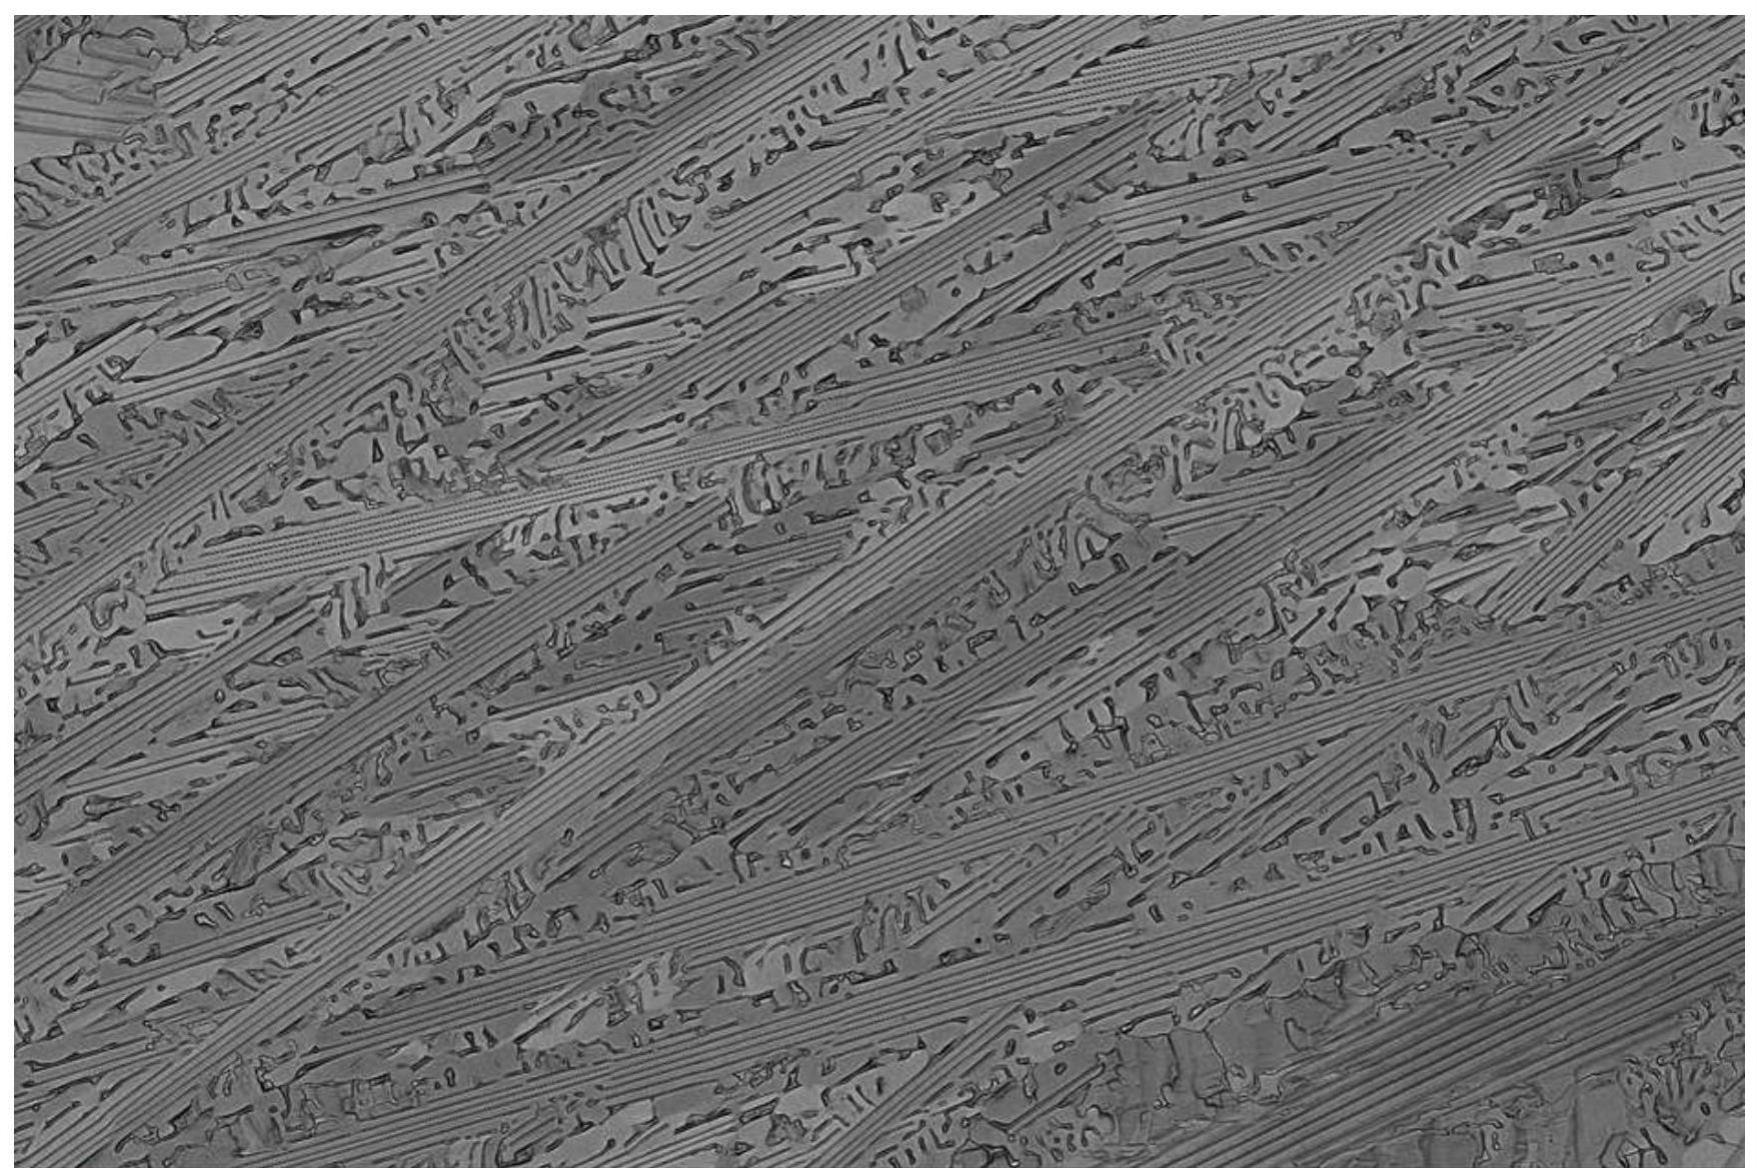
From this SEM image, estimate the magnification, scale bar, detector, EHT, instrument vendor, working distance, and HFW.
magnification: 1 K X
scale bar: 100000 nm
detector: CBS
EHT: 15 kV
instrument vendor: Thermo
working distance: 7.8 mm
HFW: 414 µm